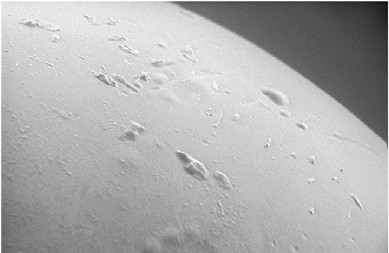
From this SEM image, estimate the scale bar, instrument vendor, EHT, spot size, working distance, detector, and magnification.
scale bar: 100000 nm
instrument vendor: FEI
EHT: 10 kV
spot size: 5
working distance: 12.2 mm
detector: GSE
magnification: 0.25 K X